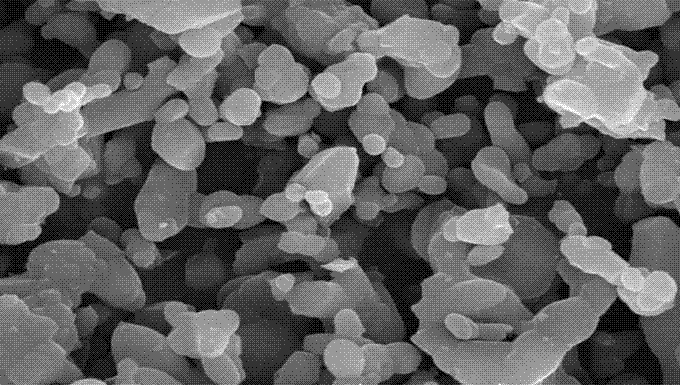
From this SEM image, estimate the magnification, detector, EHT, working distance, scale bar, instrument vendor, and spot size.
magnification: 5 K X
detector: SE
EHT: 25 kV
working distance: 5 mm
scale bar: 5000 nm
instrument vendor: FEI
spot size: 4